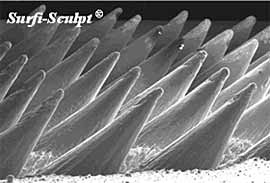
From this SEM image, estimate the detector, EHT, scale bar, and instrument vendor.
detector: SE1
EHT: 25 kV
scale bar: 1000000 nm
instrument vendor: JEOL